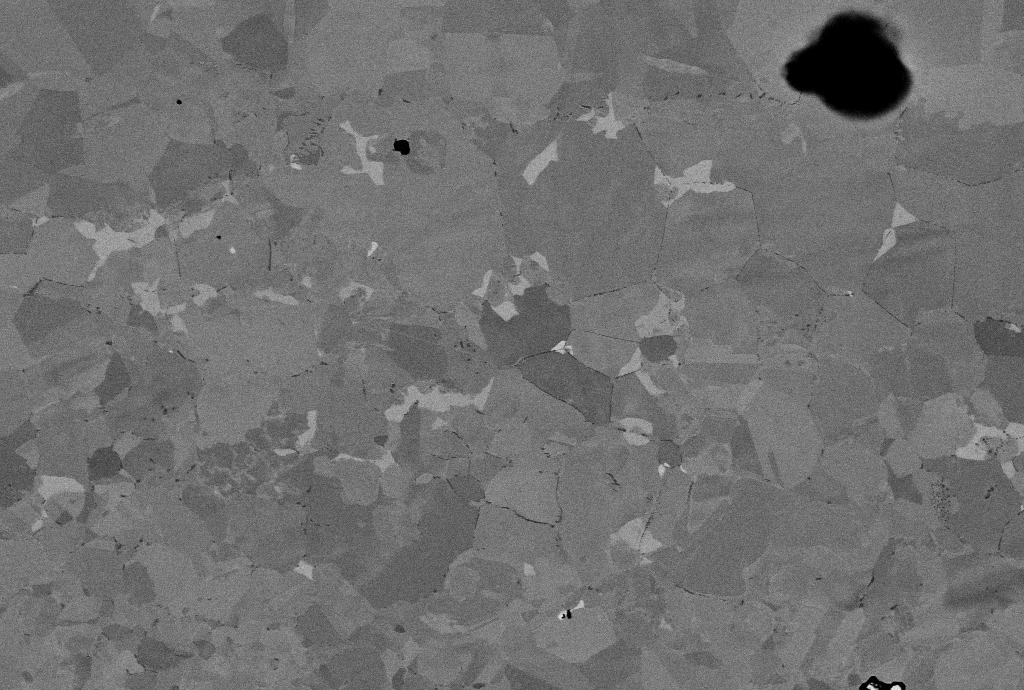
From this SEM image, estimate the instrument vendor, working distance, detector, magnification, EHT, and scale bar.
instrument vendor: Zeiss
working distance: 9 mm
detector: QBSD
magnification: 2.05 K X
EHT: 12.95 kV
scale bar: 10000 nm